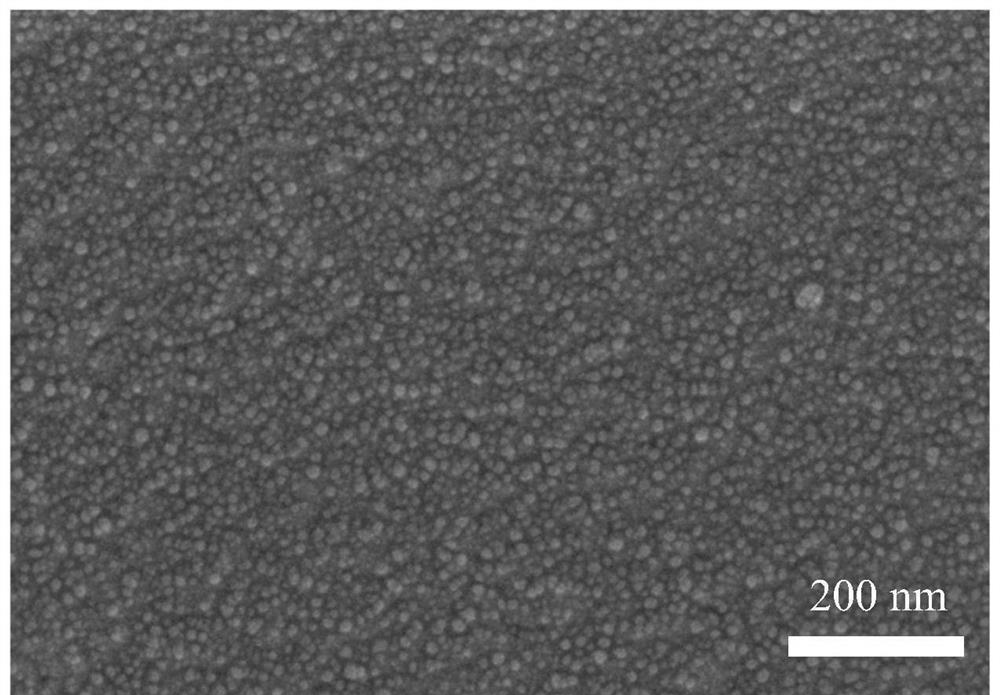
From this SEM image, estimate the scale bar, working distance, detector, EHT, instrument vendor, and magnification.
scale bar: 100 nm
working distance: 4.2 mm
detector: InLens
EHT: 5 kV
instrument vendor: Zeiss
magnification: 100 K X